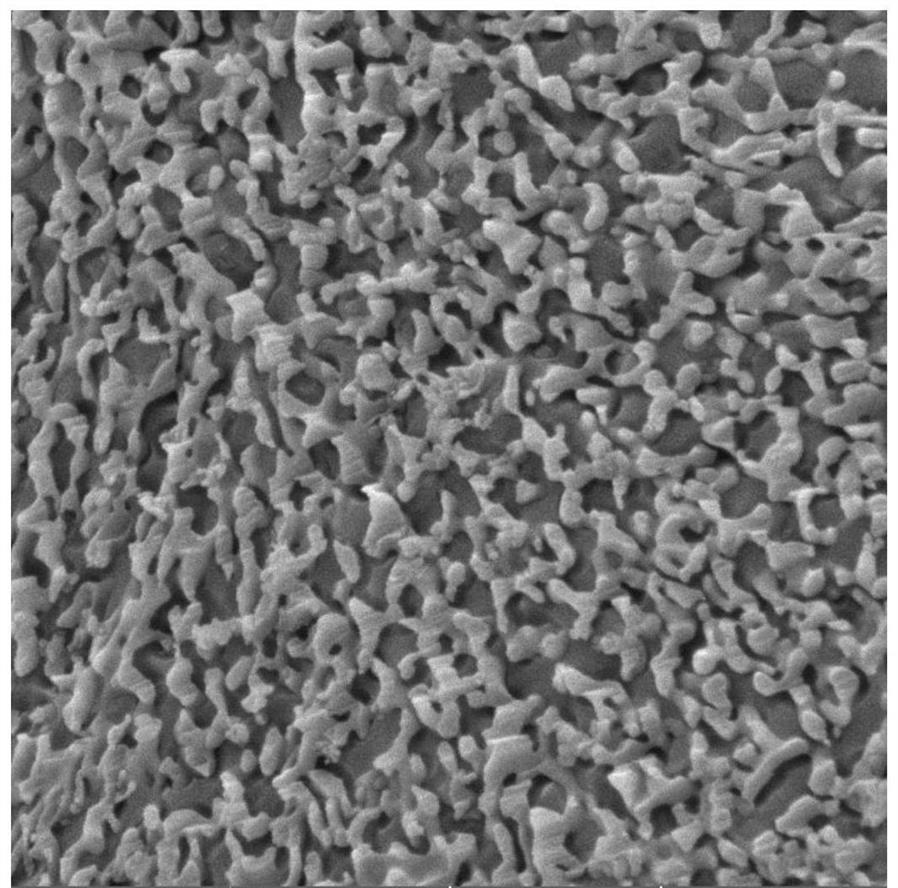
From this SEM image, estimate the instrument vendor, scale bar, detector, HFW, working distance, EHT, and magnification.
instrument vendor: Tescan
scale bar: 1000 nm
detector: SE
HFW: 4.15 µm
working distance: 7.17 mm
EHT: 5 kV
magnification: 50 K X